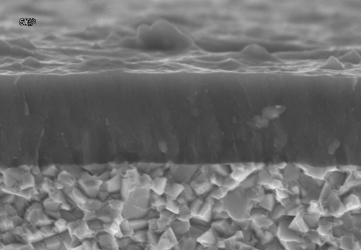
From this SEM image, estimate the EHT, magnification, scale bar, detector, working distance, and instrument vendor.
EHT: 15 kV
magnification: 10 K X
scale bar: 5000 nm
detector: SE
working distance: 6.1 mm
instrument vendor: Hitachi